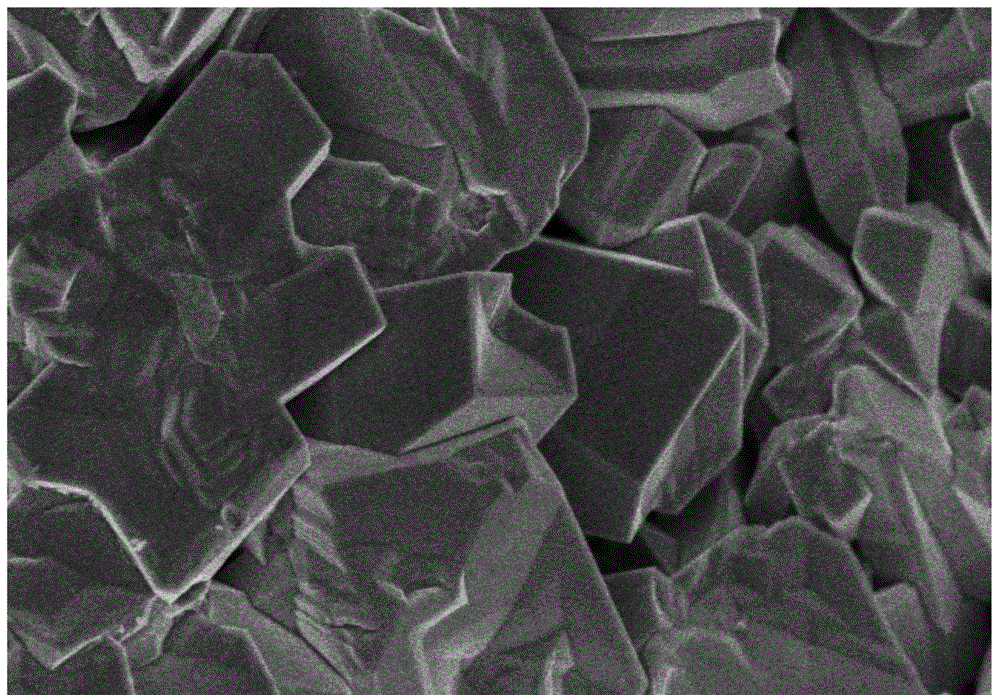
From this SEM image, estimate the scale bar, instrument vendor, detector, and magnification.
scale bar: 2000 nm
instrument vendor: Hitachi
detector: SE(U)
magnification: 25 K X